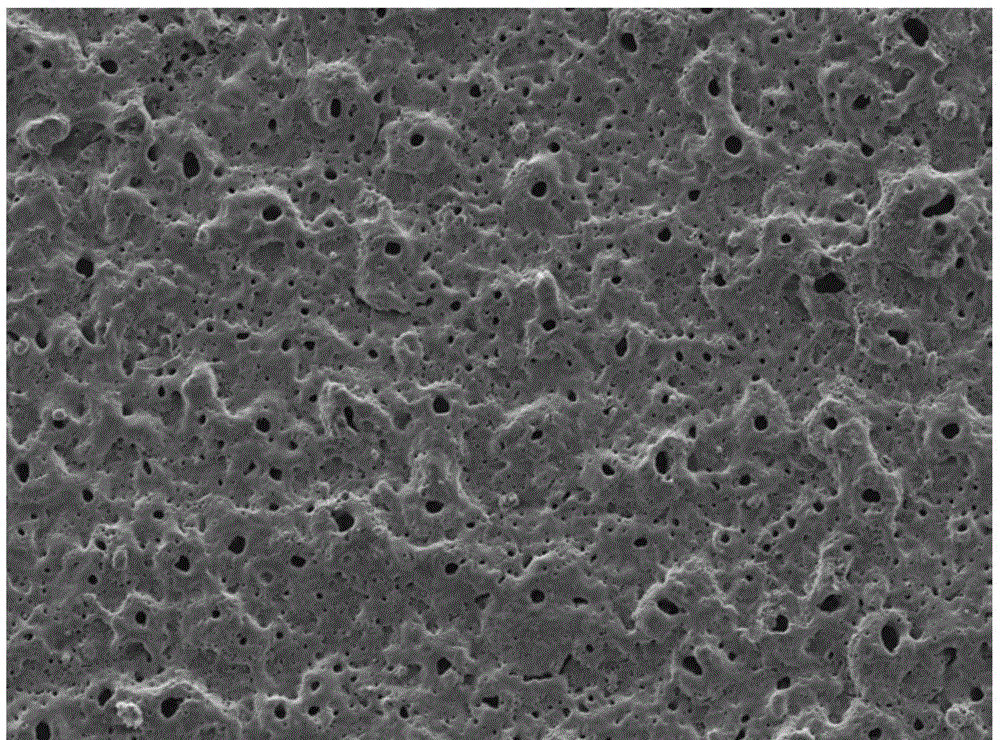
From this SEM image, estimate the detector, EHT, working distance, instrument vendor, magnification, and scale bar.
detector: SEI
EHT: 12 kV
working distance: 8 mm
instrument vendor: JEOL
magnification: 0.2 K X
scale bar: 100000 nm